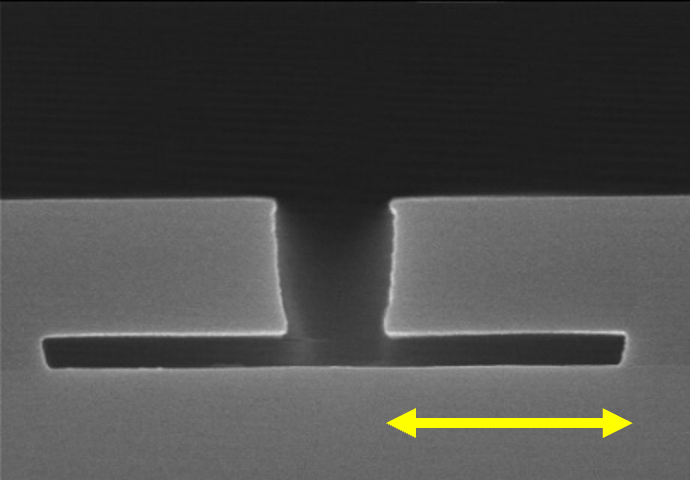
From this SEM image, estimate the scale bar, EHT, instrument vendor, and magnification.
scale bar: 1000 nm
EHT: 15 kV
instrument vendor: Hitachi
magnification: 30 K X